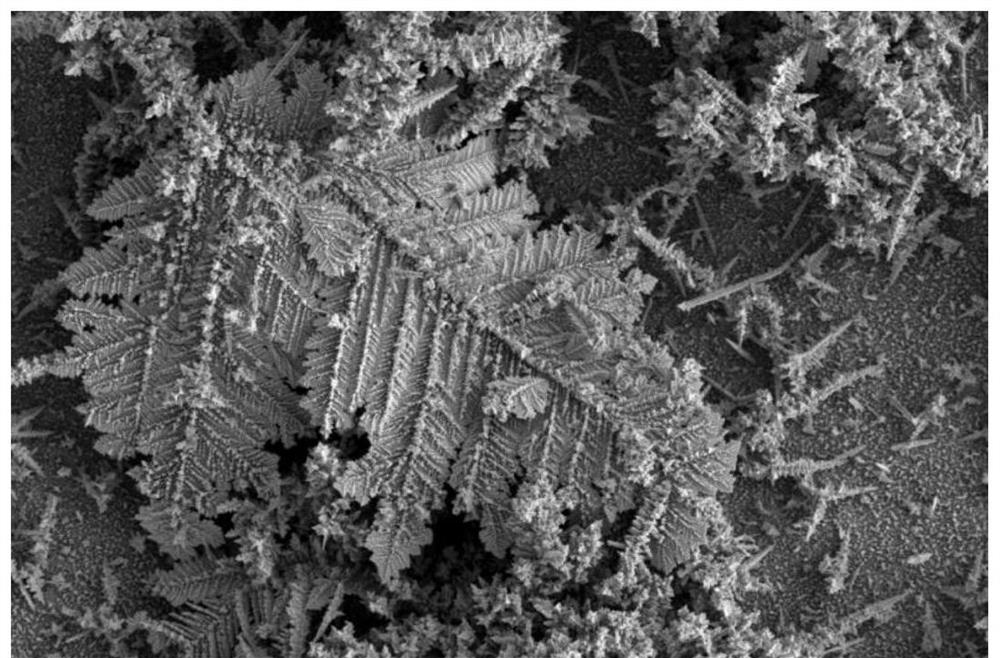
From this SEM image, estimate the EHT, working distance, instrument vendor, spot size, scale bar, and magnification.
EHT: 5 kV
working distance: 6.7 mm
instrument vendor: FEI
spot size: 3.5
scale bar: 20000 nm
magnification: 3.5 K X